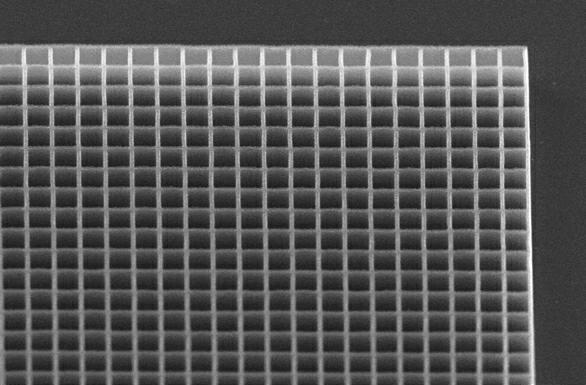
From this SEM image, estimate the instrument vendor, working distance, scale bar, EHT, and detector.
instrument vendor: Zeiss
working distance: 6 mm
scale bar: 20000 nm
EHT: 10 kV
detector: SE1